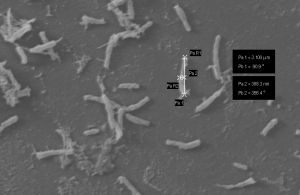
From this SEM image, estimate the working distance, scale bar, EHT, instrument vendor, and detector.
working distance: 8 mm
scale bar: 2000 nm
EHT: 20 kV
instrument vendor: Zeiss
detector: SE1